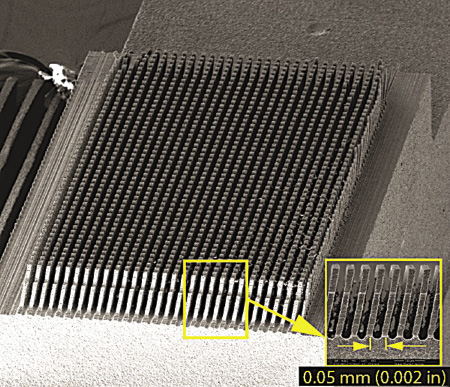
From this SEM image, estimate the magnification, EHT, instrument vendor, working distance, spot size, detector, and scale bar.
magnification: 0.13 K X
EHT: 15 kV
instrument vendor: FEI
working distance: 53.4 mm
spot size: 4.5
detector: ETD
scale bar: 1000000 nm